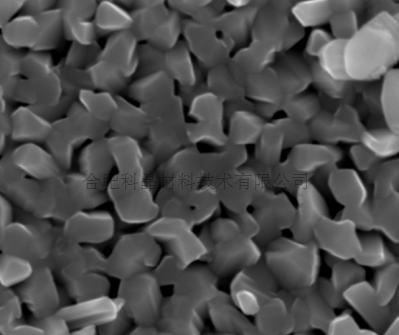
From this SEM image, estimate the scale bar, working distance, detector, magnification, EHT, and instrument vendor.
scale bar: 1000 nm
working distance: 6 mm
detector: TLD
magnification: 40 K X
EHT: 20 kV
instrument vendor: FEI